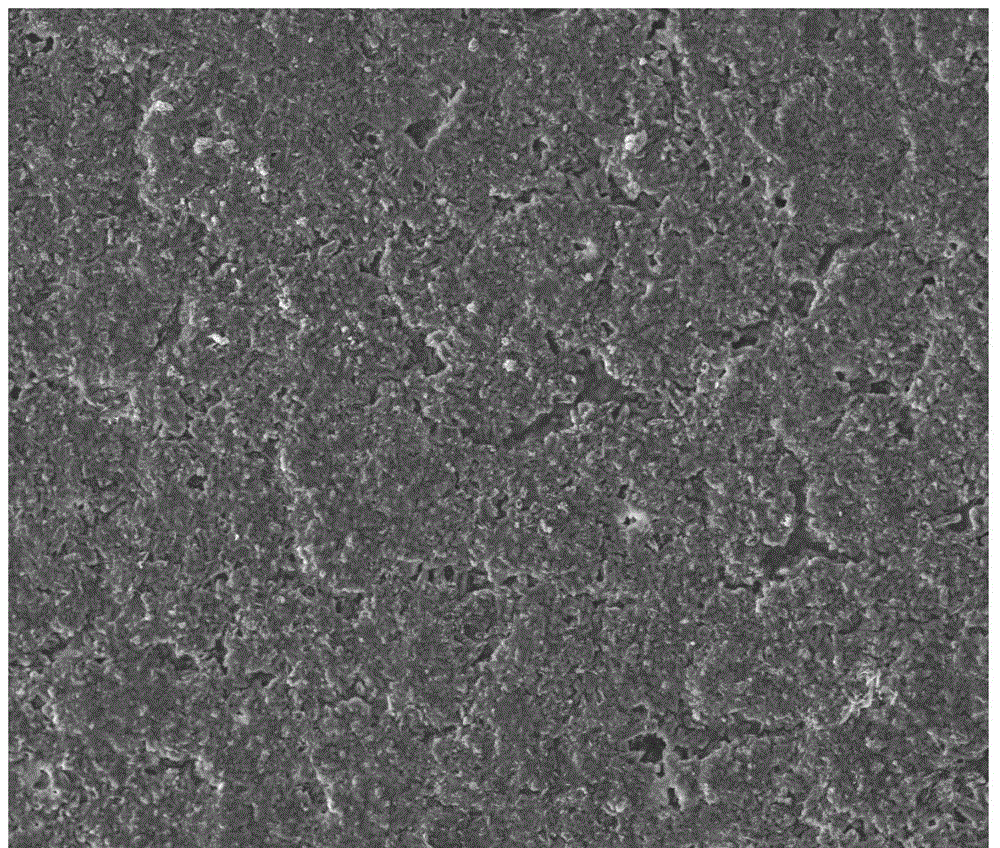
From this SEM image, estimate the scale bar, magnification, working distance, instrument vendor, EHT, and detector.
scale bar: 200000 nm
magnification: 0.5 K X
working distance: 10.8 mm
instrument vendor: FEI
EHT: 25 kV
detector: SE